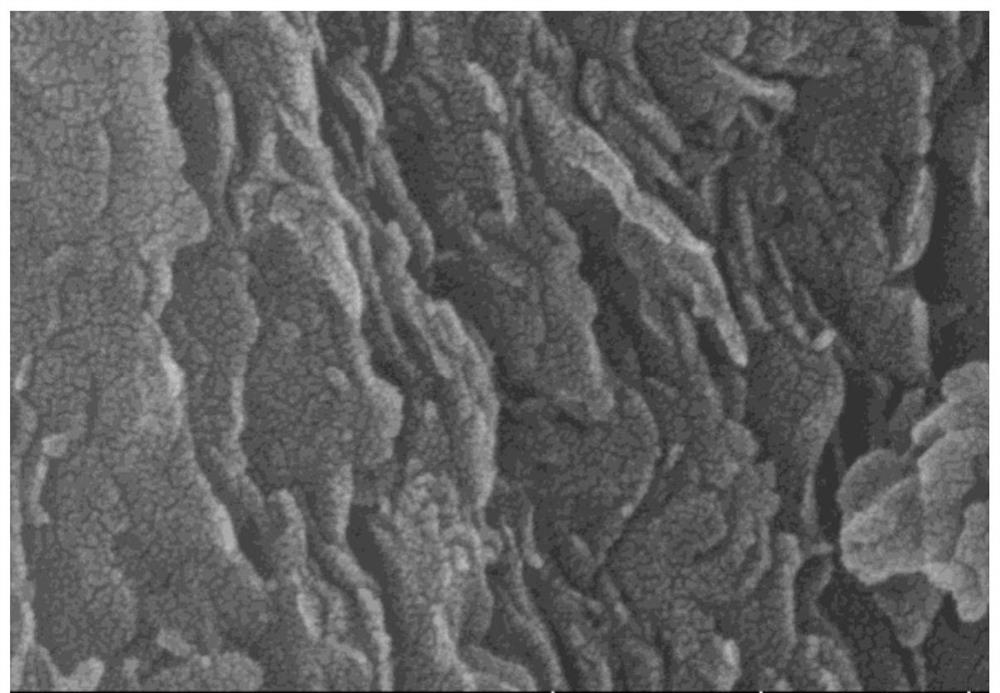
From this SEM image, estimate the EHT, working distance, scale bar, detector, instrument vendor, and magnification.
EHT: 10 kV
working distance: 8.3 mm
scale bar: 300 nm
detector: SE(UL)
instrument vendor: Hitachi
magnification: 180 K X